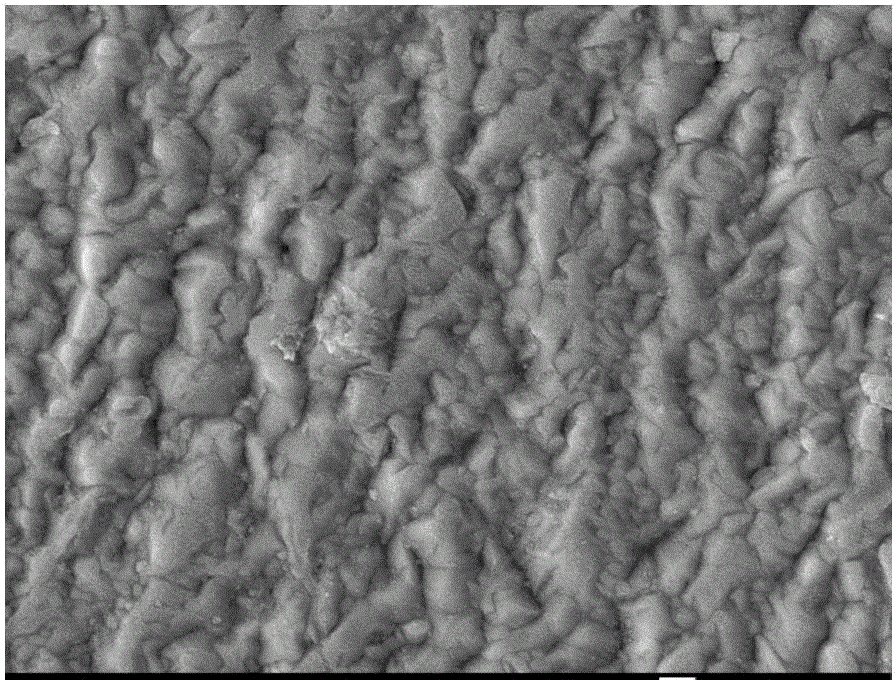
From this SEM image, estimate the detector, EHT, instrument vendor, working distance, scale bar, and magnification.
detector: LEI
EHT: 20 kV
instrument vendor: JEOL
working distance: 15.1 mm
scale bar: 1000 nm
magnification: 5 K X